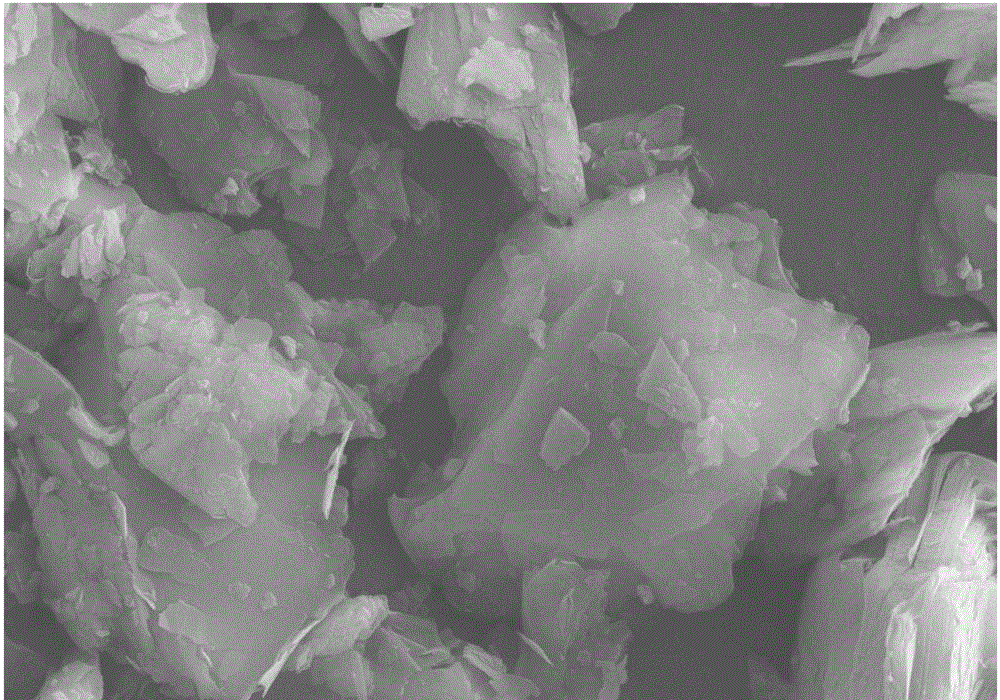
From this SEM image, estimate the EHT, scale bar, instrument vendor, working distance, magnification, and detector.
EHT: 15 kV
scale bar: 2000 nm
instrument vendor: Zeiss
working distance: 6.2 mm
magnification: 5 K X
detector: SE2